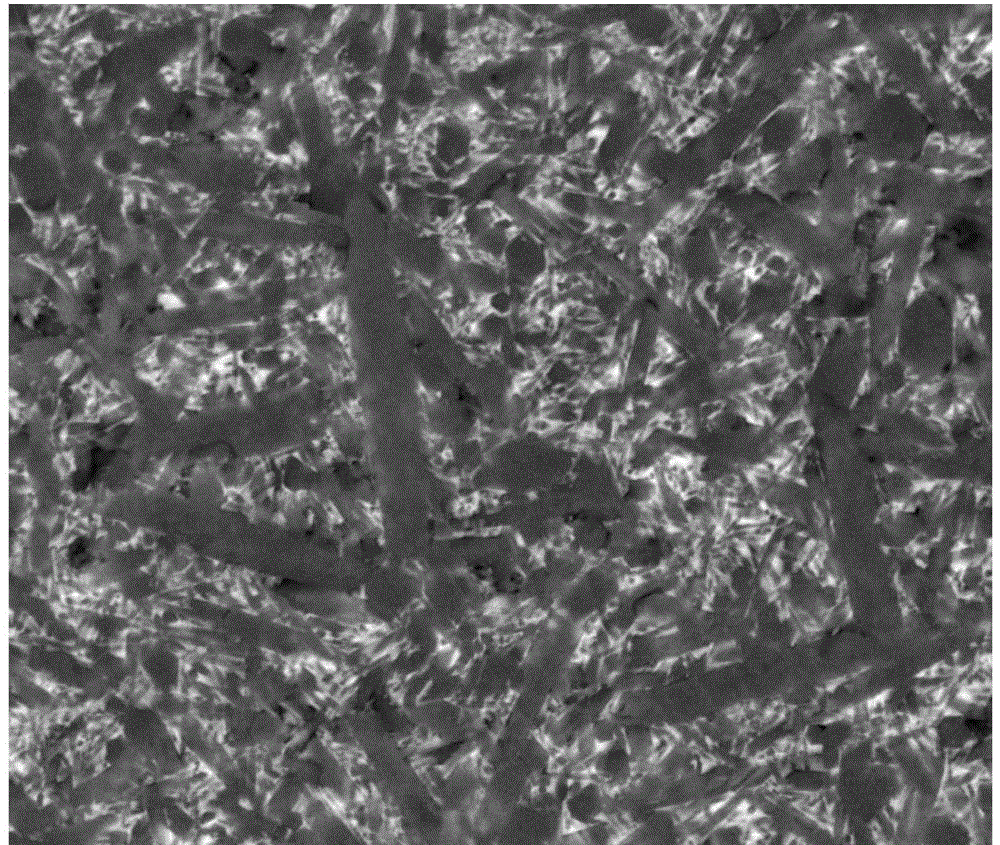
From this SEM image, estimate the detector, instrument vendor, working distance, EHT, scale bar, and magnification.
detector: BSED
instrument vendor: FEI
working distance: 6.4 mm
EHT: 20 kV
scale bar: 4000 nm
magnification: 10 K X